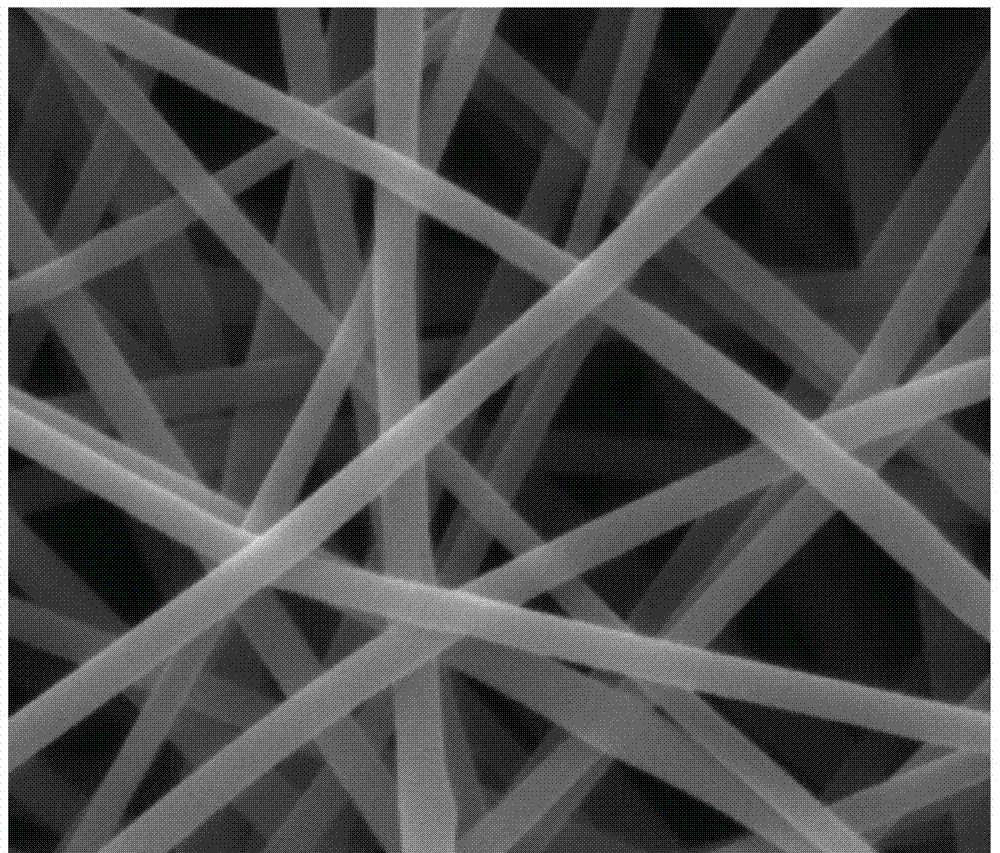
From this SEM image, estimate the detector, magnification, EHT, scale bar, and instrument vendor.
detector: ETD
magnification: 10 K X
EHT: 10 kV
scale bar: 5000 nm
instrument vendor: FEI